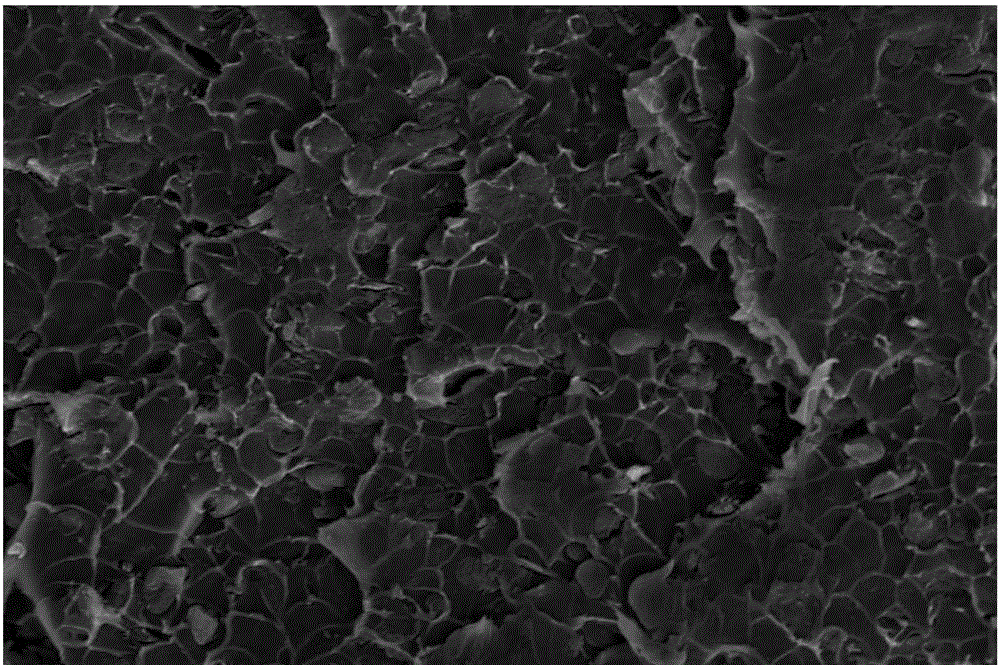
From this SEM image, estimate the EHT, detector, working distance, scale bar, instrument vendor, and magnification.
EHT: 15 kV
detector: ETD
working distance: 6.5 mm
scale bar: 50000 nm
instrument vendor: FEI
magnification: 3 K X